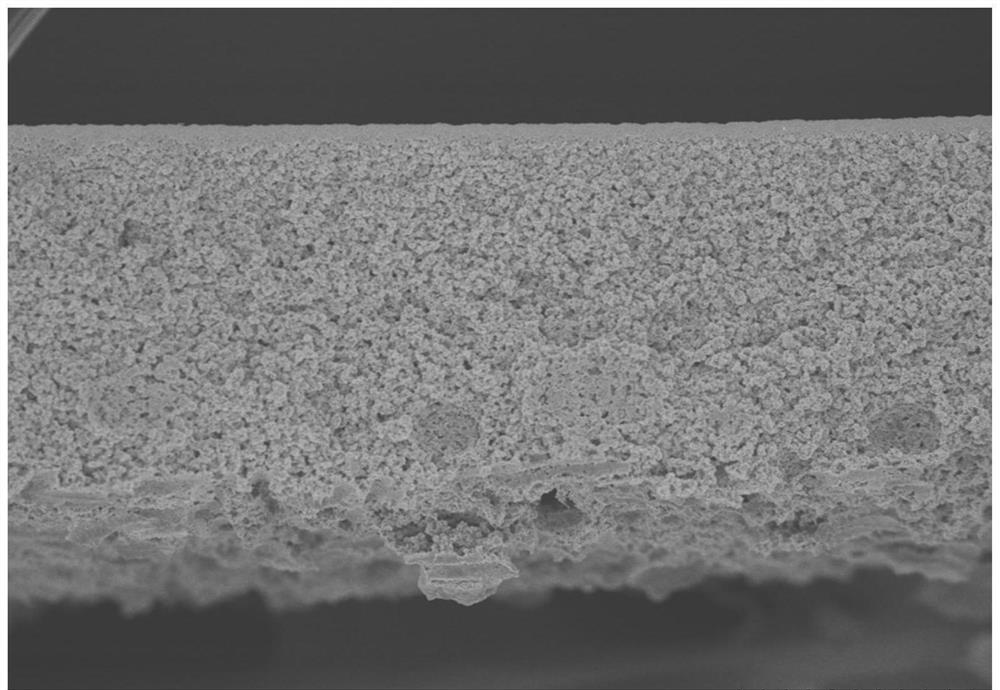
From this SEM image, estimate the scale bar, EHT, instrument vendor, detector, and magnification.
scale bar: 100000 nm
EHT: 4 kV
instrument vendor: Hitachi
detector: SE(U)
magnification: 0.3 K X